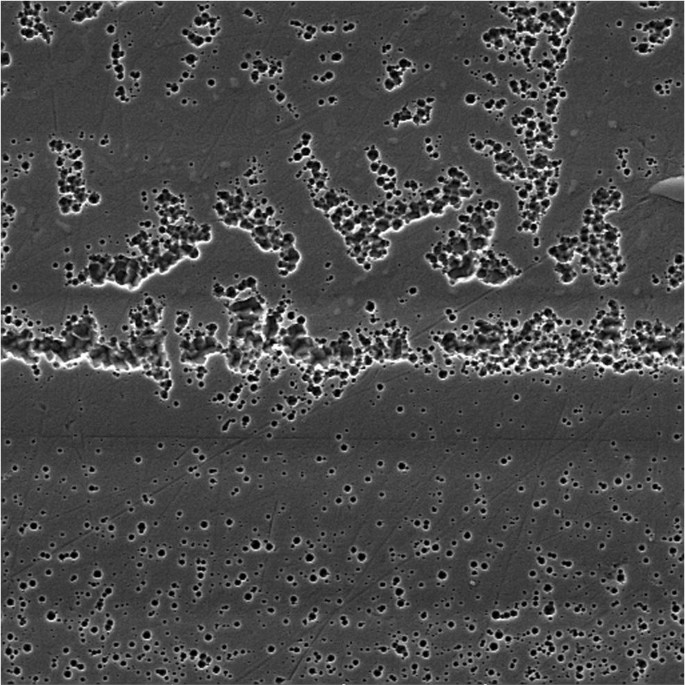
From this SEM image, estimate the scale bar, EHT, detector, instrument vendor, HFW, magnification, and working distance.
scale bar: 10000 nm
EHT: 15 kV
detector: SE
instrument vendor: Tescan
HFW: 48.2 µm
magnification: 3 K X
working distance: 11.46 mm